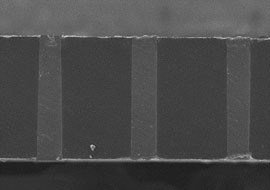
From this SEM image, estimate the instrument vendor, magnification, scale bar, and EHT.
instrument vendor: JEOL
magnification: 0.2 K X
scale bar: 150000 nm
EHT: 20 kV